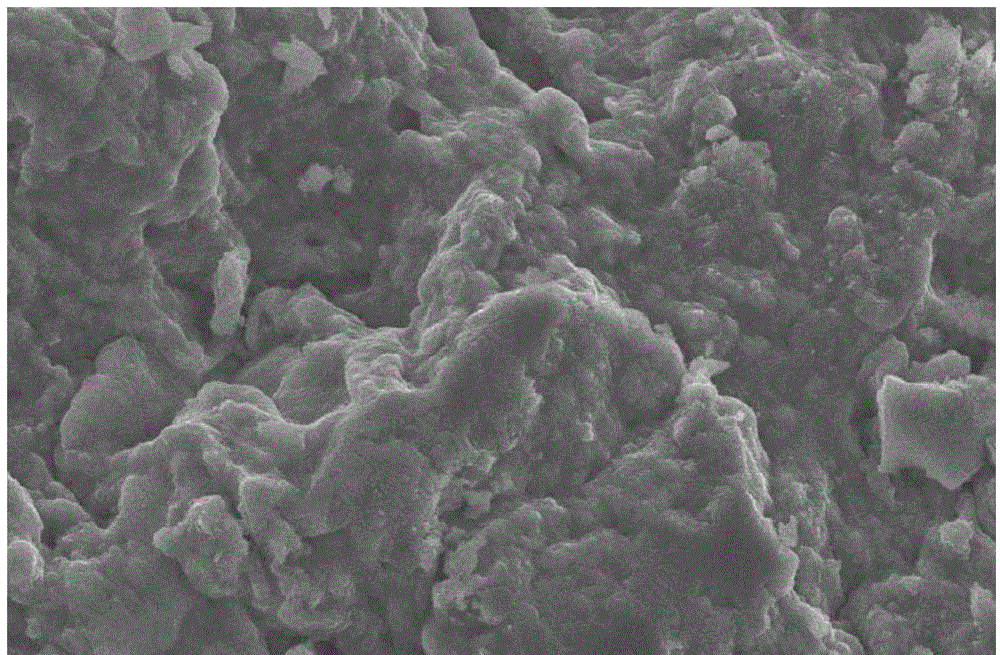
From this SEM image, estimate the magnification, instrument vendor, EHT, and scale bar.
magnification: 1 K X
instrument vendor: JEOL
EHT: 20 kV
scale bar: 10000 nm